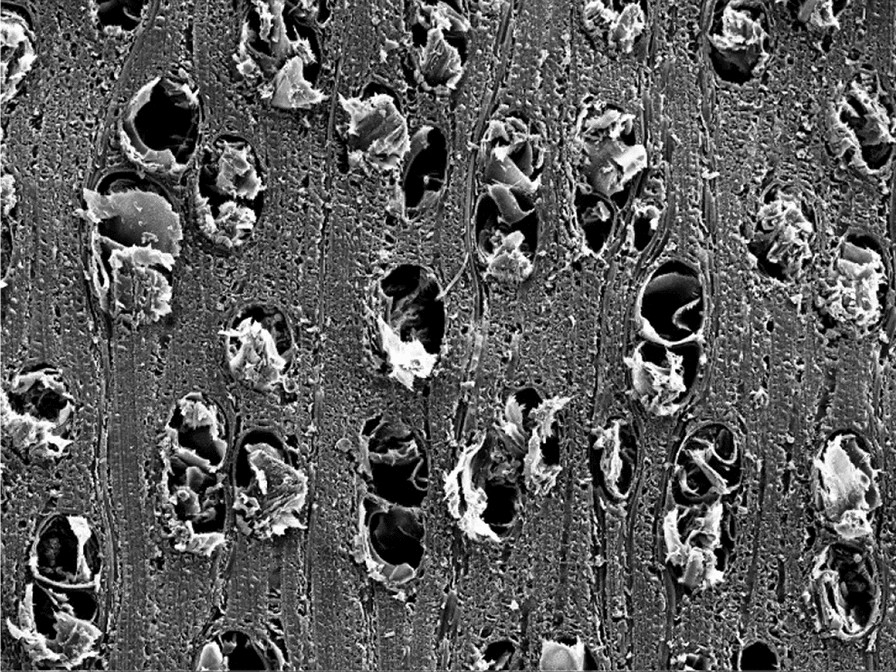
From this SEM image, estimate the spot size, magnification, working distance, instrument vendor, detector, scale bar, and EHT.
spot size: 2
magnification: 0.2 K X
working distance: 16.73 mm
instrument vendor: other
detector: SEI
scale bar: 60000 nm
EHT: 20 kV